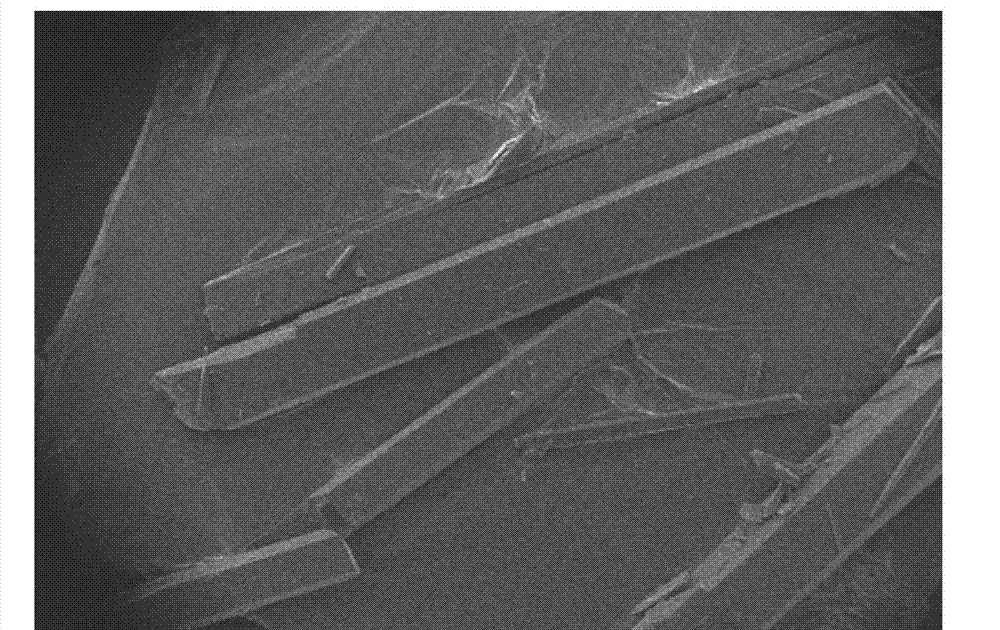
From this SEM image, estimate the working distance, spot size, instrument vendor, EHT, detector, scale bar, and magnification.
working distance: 11.5 mm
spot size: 3.2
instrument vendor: FEI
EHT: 20 kV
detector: SE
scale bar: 2000000 nm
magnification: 0.019 K X